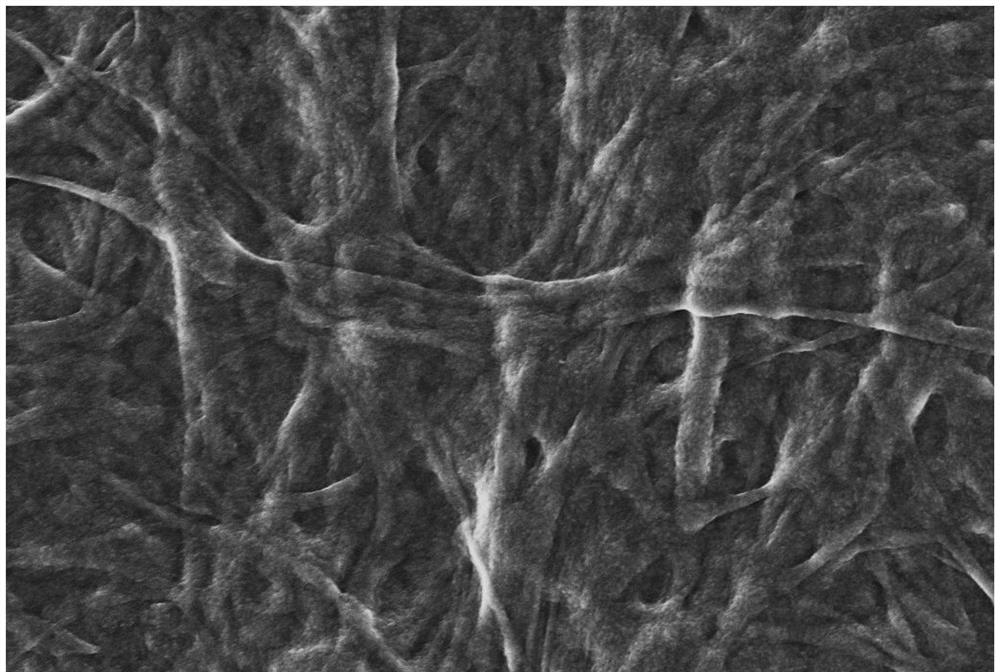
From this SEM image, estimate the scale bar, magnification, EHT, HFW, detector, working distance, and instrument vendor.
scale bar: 2000 nm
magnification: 5 K X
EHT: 5 kV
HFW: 22.87 µm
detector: InLens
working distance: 4.3 mm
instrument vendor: Zeiss